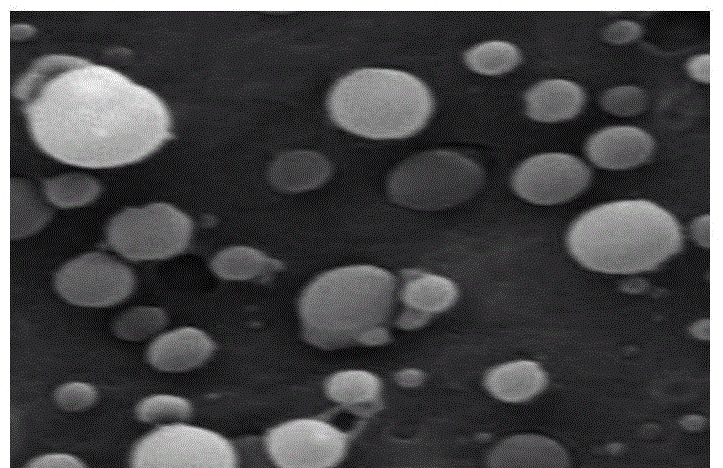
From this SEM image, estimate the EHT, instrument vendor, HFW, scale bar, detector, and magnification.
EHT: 20 kV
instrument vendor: Tescan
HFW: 15 µm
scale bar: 2000 nm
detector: SE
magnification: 10 K X